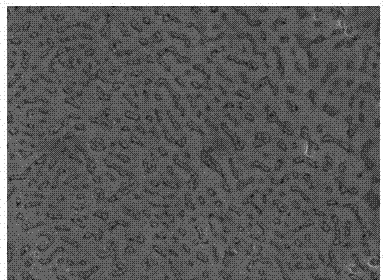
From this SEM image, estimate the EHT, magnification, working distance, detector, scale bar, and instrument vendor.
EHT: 20 kV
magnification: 4 K X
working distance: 9.2 mm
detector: SE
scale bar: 10000 nm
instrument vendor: Hitachi